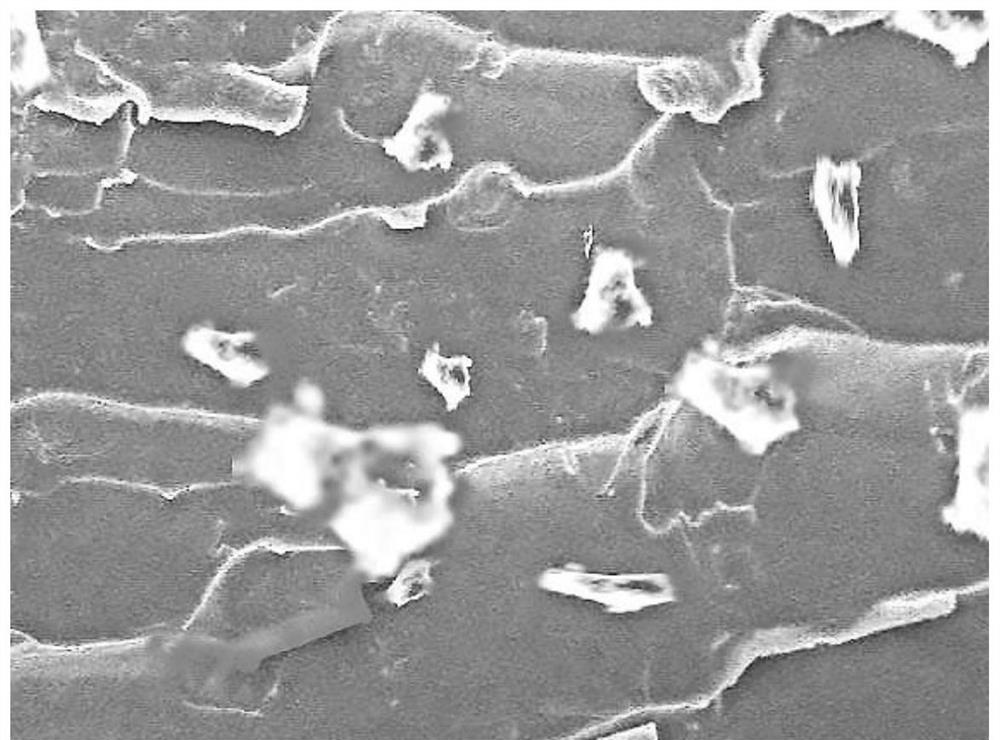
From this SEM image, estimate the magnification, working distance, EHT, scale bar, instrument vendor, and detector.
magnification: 5 K X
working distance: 9 mm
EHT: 3 kV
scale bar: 1000 nm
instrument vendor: Zeiss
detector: SE2